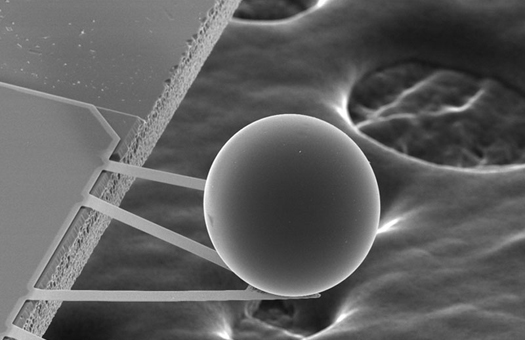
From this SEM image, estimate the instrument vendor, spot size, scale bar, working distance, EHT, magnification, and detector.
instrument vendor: FEI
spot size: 3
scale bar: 100000 nm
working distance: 34.4 mm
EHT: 20 kV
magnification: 0.384 K X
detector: SE